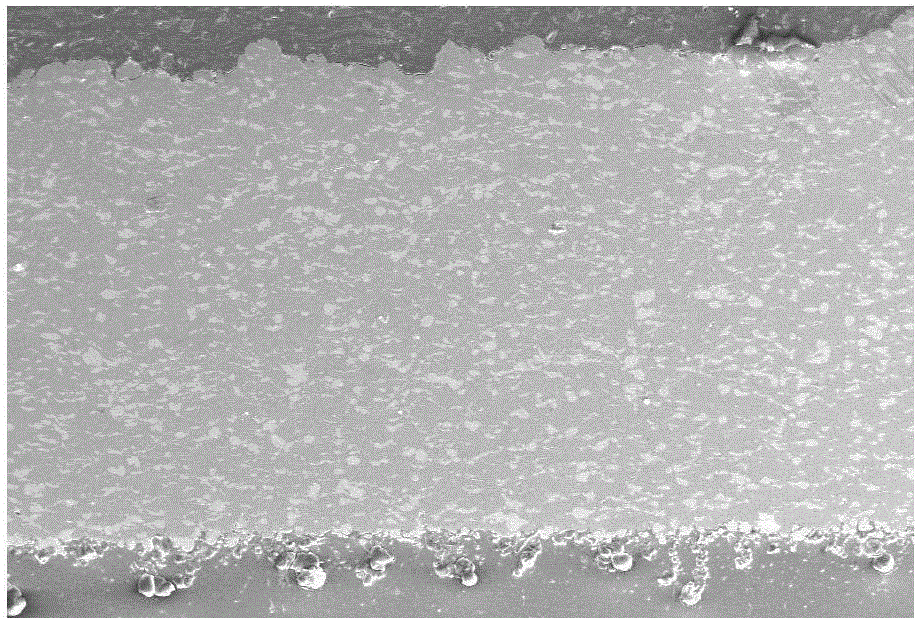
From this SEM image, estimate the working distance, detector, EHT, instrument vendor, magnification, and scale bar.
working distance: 10 mm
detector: SE1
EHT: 20 kV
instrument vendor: Zeiss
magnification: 0.074 K X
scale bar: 100000 nm